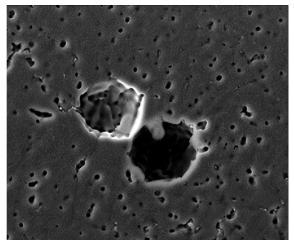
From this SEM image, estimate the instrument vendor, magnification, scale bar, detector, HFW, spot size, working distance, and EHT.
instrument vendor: FEI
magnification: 1 K X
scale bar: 50000 nm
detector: ETD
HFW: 256 µm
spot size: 4.8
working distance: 13.8 mm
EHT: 30 kV